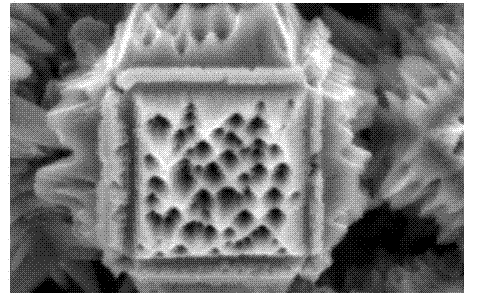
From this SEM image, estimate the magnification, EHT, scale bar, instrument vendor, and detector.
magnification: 16 K X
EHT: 10 kV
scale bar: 1000 nm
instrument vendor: JEOL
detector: SEI